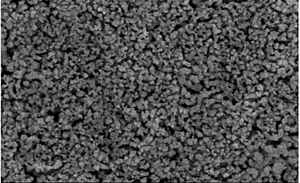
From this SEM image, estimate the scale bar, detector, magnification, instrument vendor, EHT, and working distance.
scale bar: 2000 nm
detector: SE(M)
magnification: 20 K X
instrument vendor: Hitachi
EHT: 15 kV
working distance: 12.9 mm